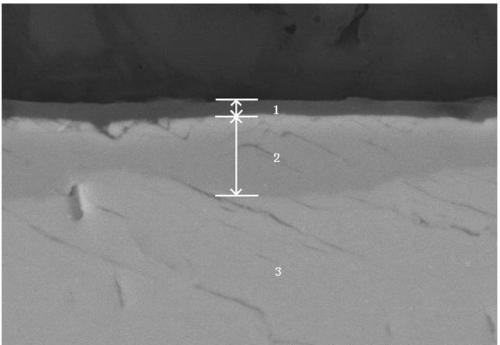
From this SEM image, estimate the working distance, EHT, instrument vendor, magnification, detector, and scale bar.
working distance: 15 mm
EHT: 20 kV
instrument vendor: Hitachi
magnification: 5 K X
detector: SE(M,LA100)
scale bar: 10000 nm